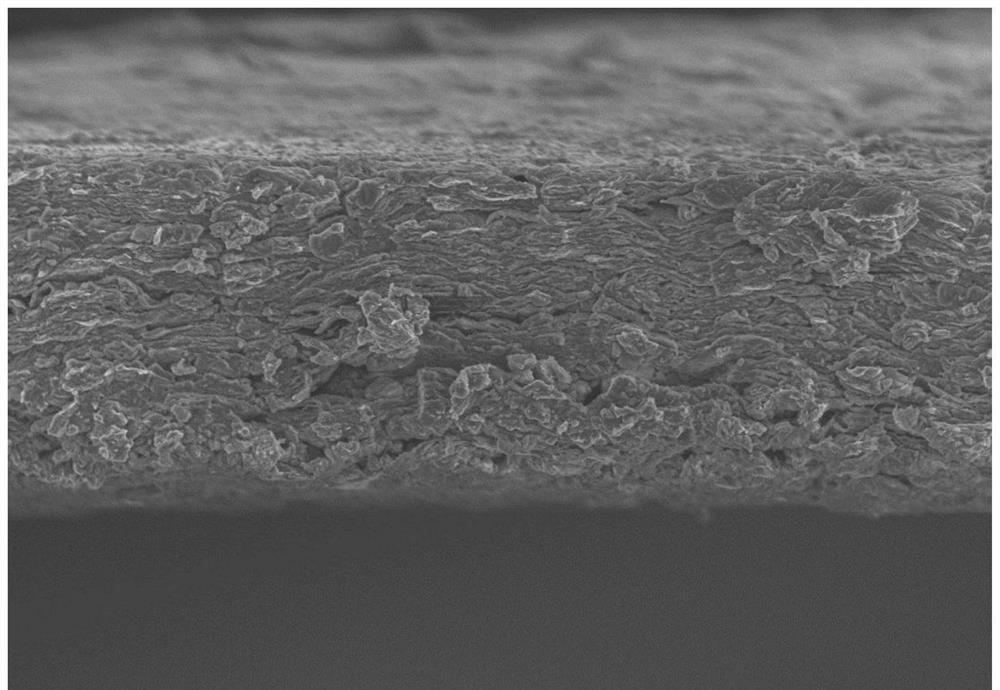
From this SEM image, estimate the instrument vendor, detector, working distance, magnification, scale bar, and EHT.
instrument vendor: Hitachi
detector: SE(M)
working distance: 6.1 mm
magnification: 1 K X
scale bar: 50000 nm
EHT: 5 kV